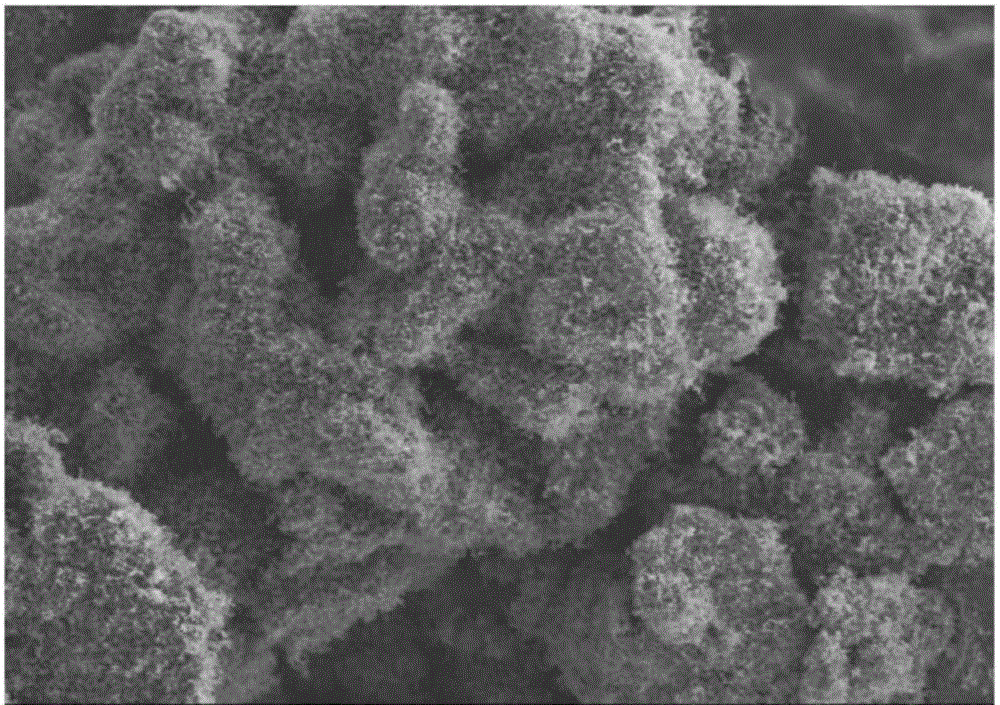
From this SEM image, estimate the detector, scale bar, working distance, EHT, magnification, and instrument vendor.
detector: SE(M)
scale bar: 20000 nm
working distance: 9.6 mm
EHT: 5 kV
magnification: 2 K X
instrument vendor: Hitachi